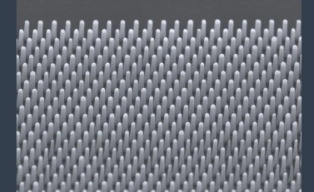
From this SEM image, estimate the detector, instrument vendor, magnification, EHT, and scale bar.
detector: SE(UL)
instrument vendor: Hitachi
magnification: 30 K X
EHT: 4 kV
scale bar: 1000 nm